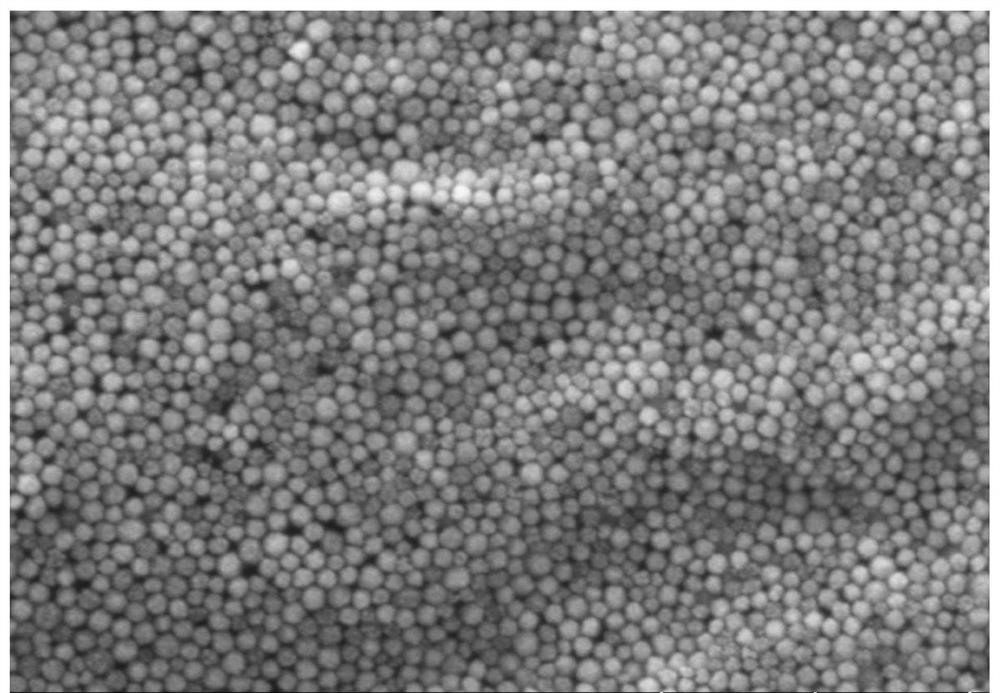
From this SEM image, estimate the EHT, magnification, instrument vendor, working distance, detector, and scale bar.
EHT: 5 kV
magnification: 20 K X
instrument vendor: Hitachi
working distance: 12.4 mm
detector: SE(TUL)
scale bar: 2000 nm